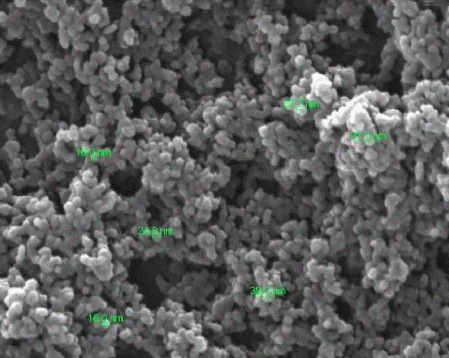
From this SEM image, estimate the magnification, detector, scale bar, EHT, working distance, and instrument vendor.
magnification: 200 K X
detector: SE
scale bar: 500 nm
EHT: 15 kV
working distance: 6.3 mm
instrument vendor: FEI